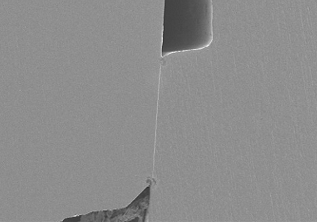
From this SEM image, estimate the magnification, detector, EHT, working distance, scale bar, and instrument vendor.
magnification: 0.1 K X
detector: SE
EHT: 10 kV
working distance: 14.8 mm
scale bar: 500000 nm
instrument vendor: Hitachi